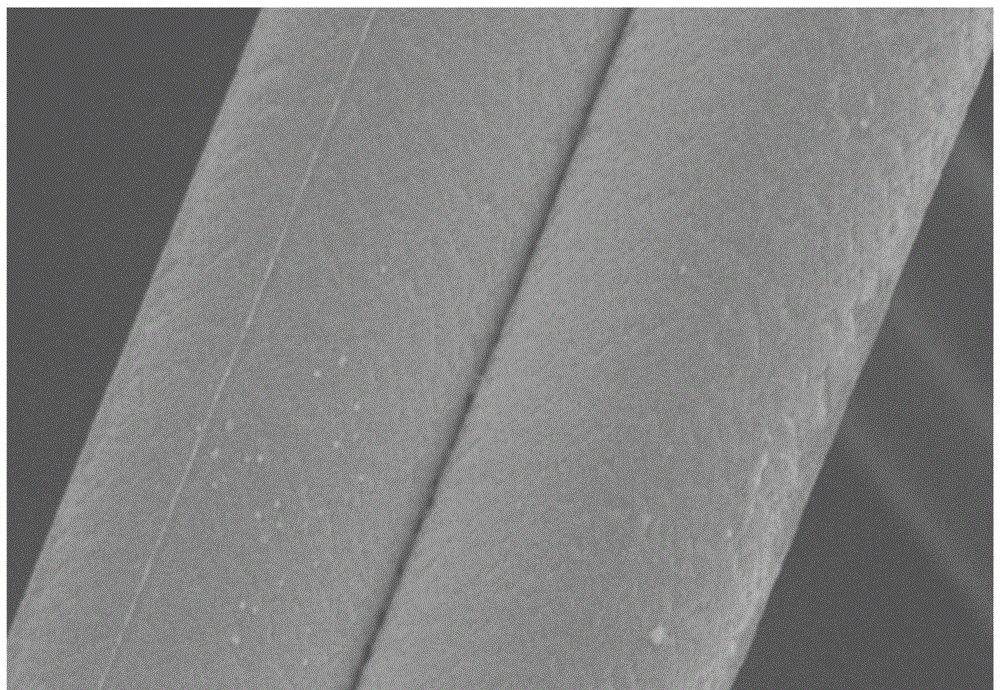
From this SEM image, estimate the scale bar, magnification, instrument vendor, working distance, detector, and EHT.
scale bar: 10000 nm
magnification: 4 K X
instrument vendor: Hitachi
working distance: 10.6 mm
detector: SE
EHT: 5 kV